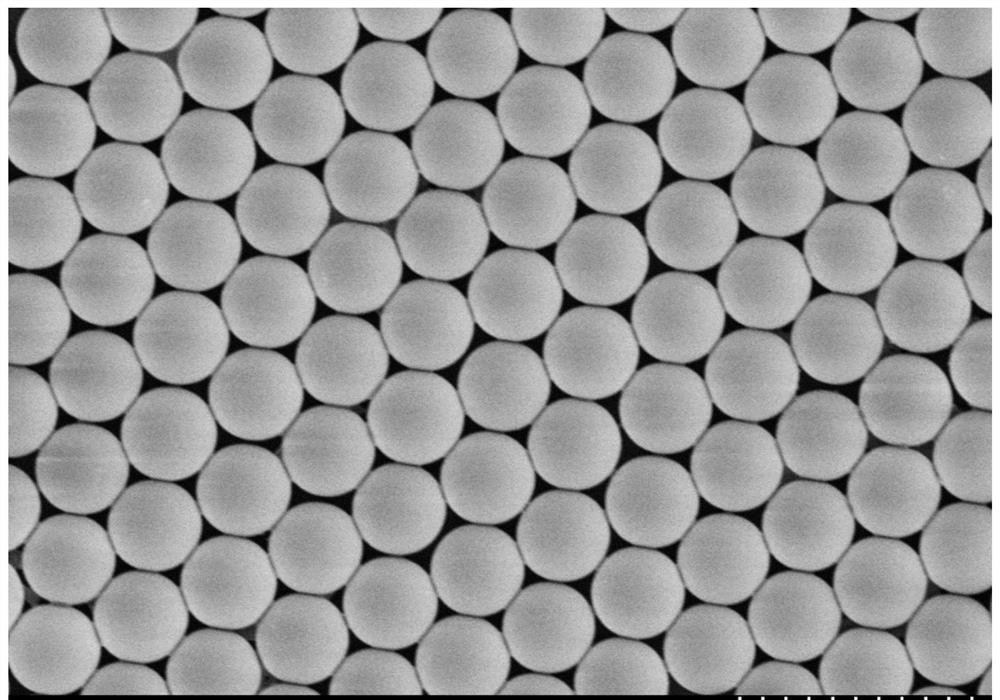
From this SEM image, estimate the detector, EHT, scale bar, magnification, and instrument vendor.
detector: SE(M)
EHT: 8 kV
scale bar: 5000 nm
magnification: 6 K X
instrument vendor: Hitachi